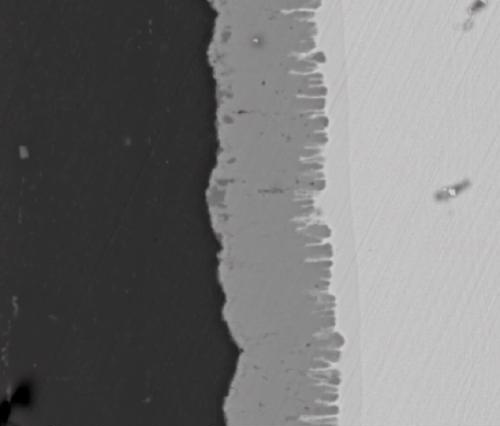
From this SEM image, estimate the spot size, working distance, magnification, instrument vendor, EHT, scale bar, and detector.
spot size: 6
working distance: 10.8 mm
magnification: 5 K X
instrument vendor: FEI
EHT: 20 kV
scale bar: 20000 nm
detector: DualBSD